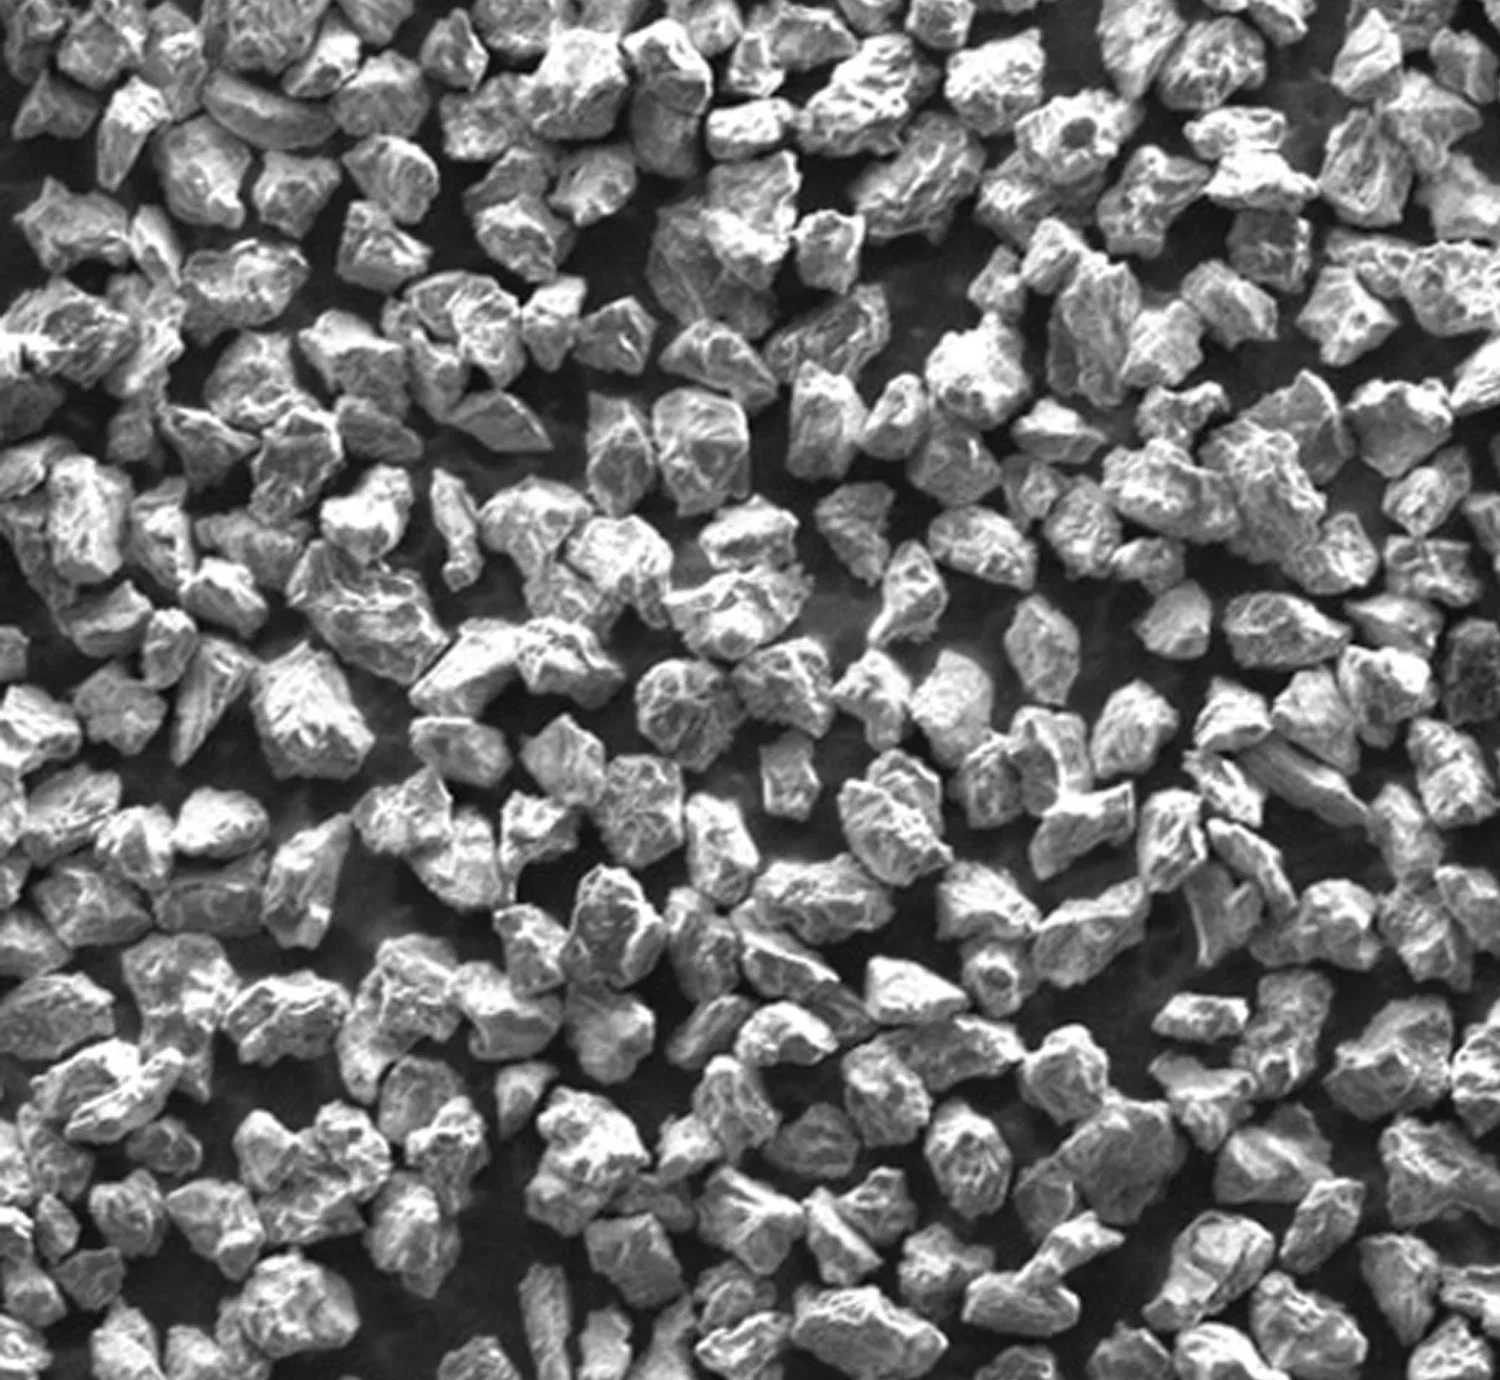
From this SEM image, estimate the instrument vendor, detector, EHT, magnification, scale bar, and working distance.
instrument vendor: Hitachi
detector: SE(M)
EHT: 5 kV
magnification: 1 K X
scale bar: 50000 nm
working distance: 9.8 mm